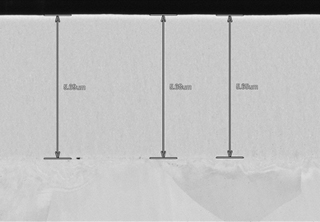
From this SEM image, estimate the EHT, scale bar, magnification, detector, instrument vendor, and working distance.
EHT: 10 kV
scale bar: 5000 nm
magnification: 10 K X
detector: LA50(UL)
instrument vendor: Hitachi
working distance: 8.2 mm